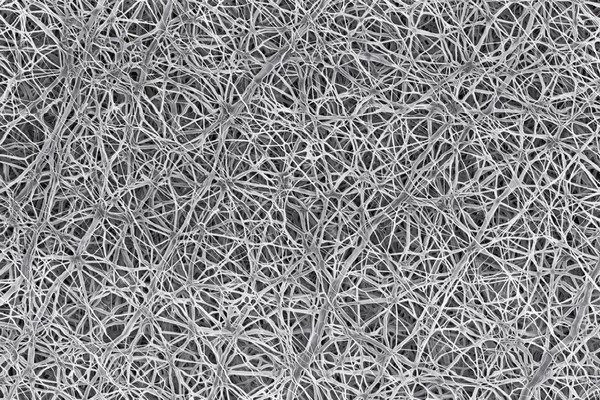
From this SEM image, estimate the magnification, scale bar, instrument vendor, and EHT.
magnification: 5 K X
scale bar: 1000 nm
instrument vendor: Zeiss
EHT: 2 kV